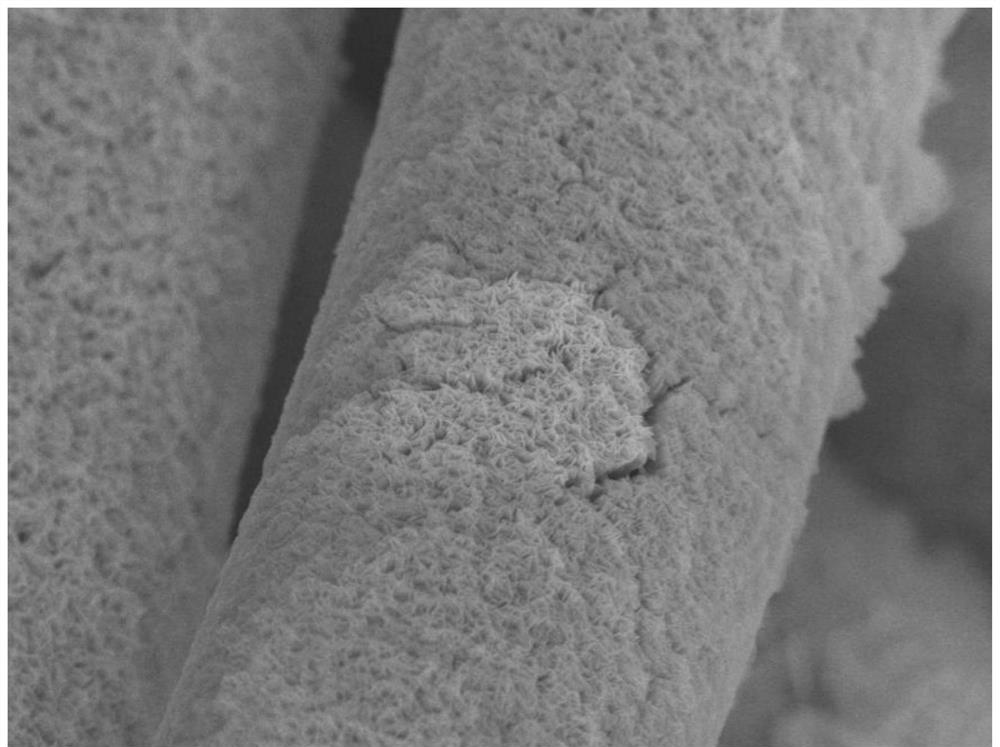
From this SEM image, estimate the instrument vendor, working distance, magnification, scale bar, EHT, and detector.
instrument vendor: JEOL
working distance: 8.9 mm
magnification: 5 K X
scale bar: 1000 nm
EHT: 8 kV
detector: SEI